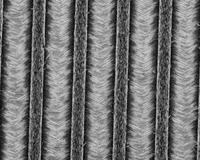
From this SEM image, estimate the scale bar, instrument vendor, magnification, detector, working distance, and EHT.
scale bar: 50000 nm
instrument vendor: FEI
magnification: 1.25 K X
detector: TLD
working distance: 5.2 mm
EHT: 5 kV